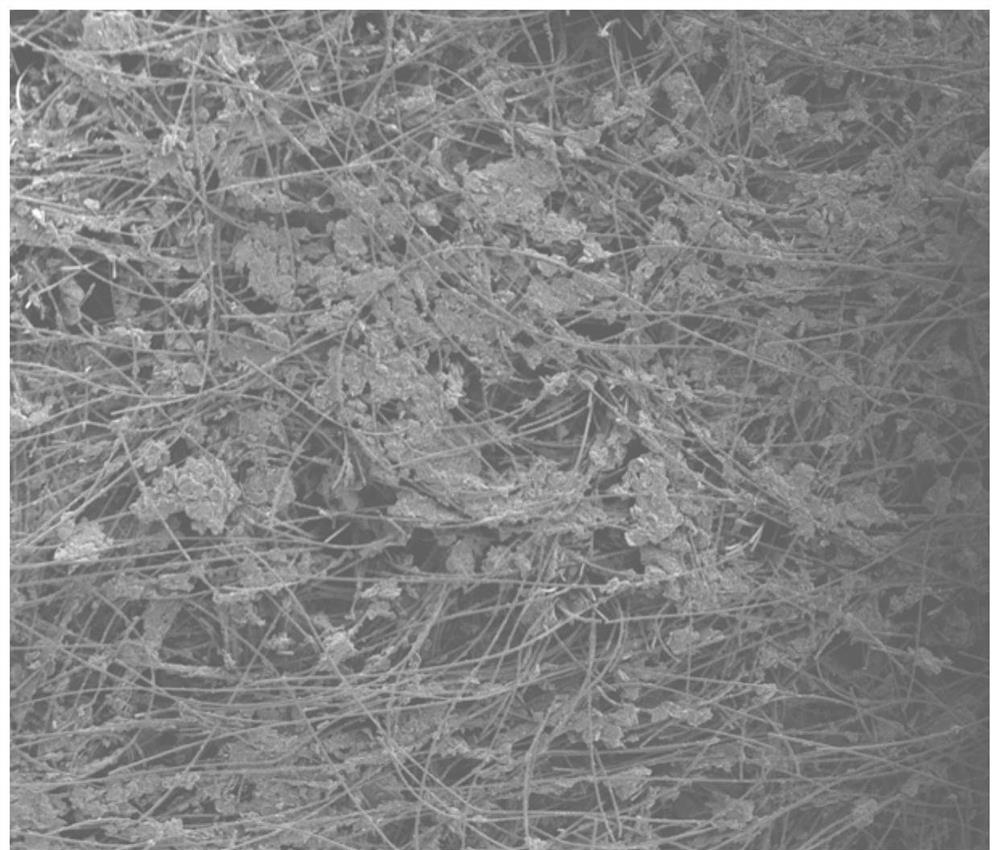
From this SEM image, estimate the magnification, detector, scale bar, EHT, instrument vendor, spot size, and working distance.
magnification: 0.1 K X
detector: ETD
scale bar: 1000000 nm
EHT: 10 kV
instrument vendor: FEI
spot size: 3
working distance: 9.9 mm